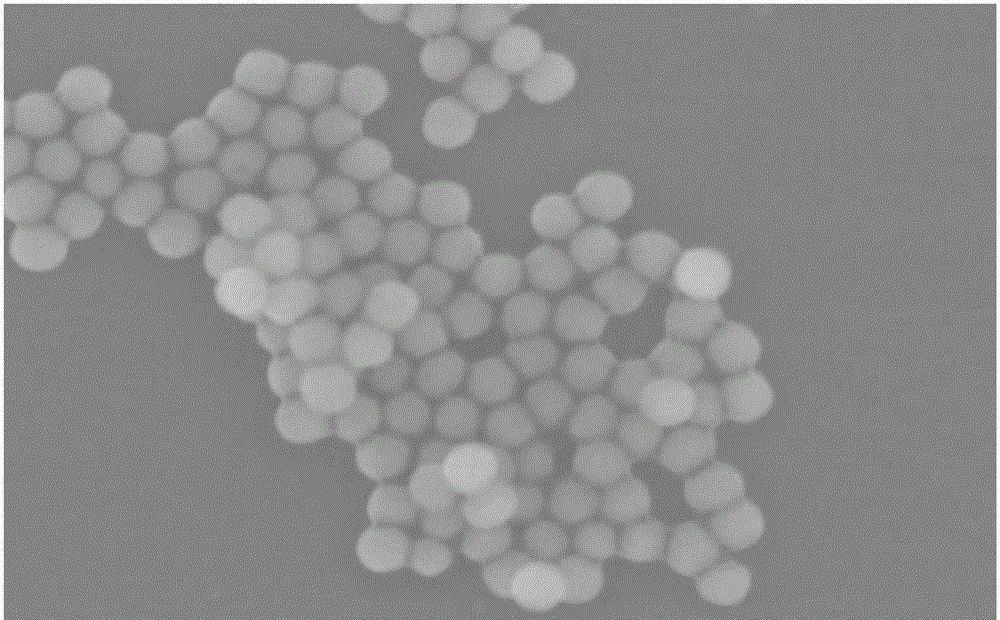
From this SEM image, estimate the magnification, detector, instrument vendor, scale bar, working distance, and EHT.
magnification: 130 K X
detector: SE(M)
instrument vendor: Hitachi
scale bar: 400 nm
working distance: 8.8 mm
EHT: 5 kV